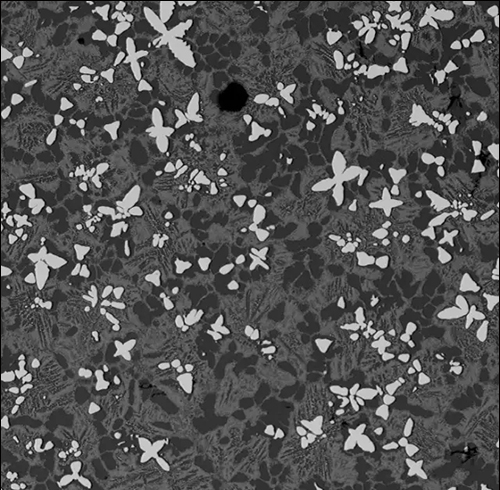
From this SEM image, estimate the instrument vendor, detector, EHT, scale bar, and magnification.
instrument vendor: Phenom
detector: BSD Full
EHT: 15 kV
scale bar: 100000 nm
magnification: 0.5 K X